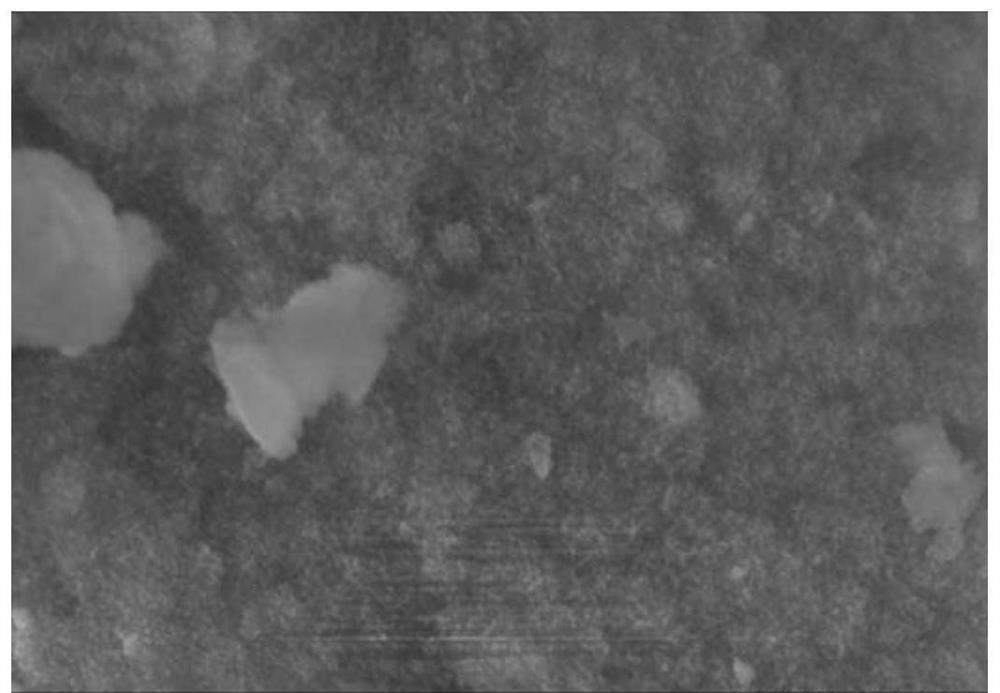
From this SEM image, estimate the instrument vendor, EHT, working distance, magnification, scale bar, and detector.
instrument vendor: Hitachi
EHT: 5 kV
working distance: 3.7 mm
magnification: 60 K X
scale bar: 500 nm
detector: SE(M)